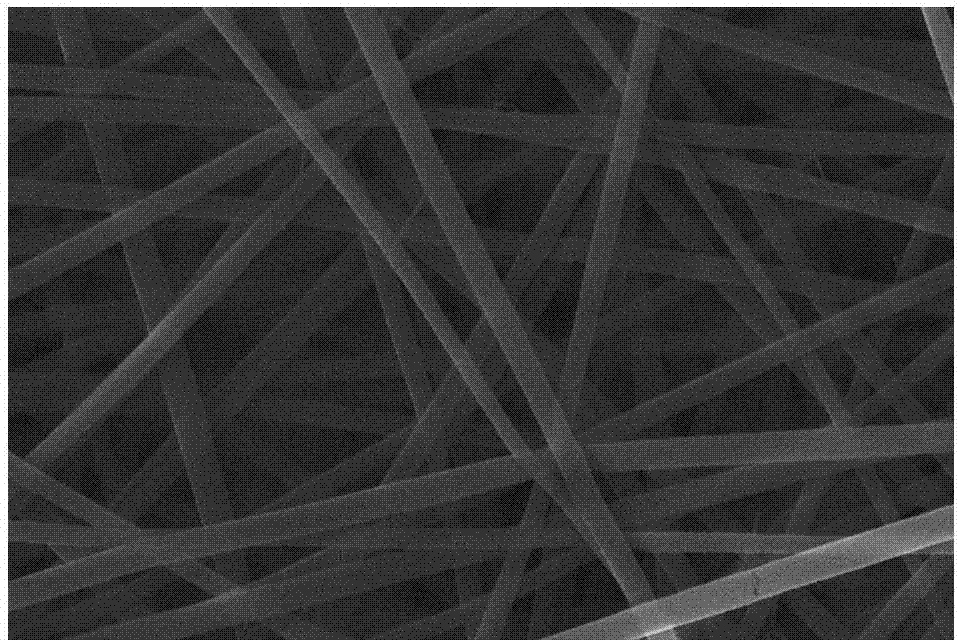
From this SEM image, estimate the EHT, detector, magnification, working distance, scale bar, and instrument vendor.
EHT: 20 kV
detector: InLens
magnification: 20 K X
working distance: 5.6 mm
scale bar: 200 nm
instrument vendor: Zeiss